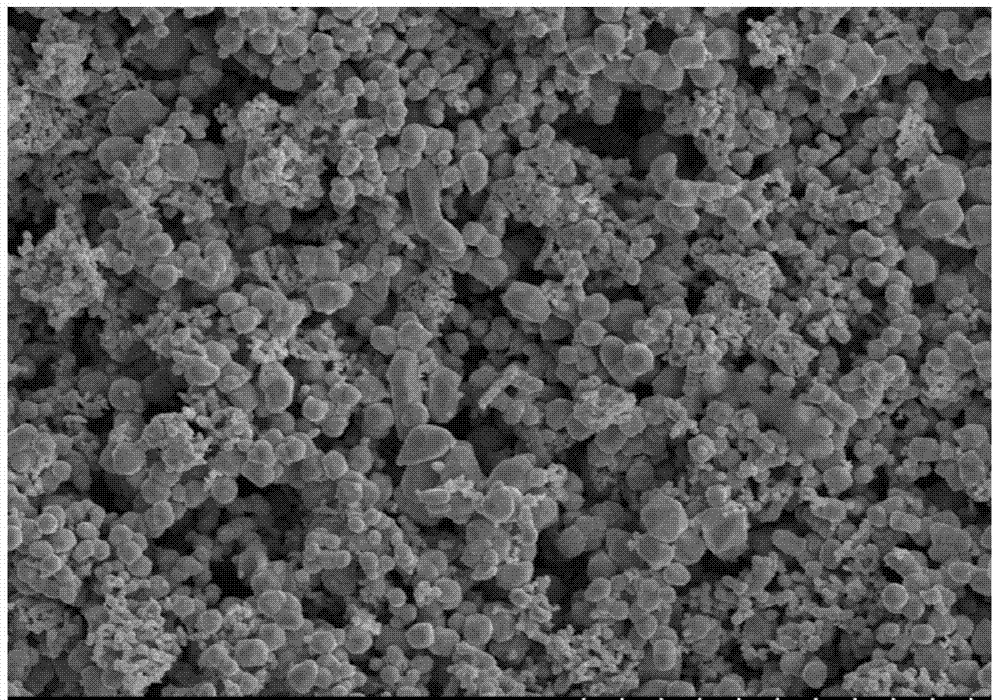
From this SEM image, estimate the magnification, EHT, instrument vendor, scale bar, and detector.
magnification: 1 K X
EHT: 20 kV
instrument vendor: Hitachi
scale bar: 50000 nm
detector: SE